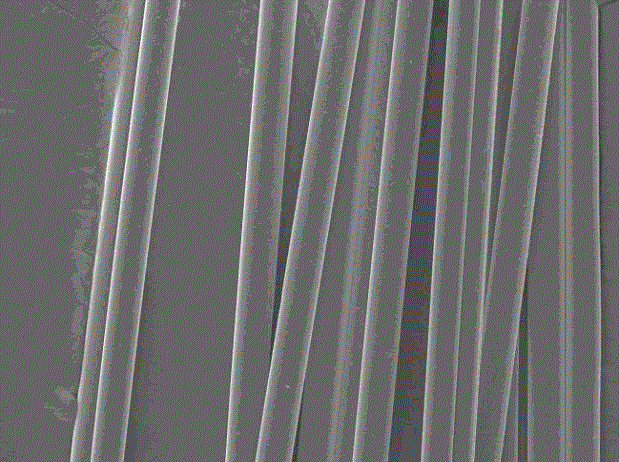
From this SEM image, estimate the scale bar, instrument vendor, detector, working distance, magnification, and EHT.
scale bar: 10000 nm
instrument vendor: JEOL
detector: SEI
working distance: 11.2 mm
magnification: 0.5 K X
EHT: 15 kV